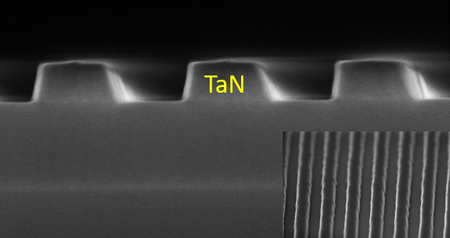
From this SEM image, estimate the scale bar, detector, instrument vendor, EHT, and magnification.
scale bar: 500 nm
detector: BE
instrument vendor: Hitachi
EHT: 5 kV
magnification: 100 K X